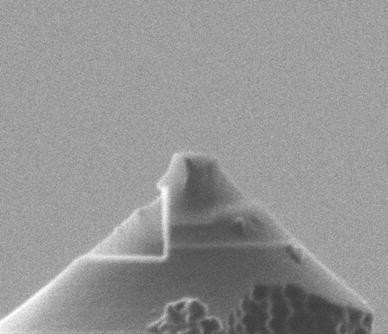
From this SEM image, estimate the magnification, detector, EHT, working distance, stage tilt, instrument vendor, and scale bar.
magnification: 65 K X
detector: SED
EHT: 30 kV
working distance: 18 mm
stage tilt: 10°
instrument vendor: FEI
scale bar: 1000 nm